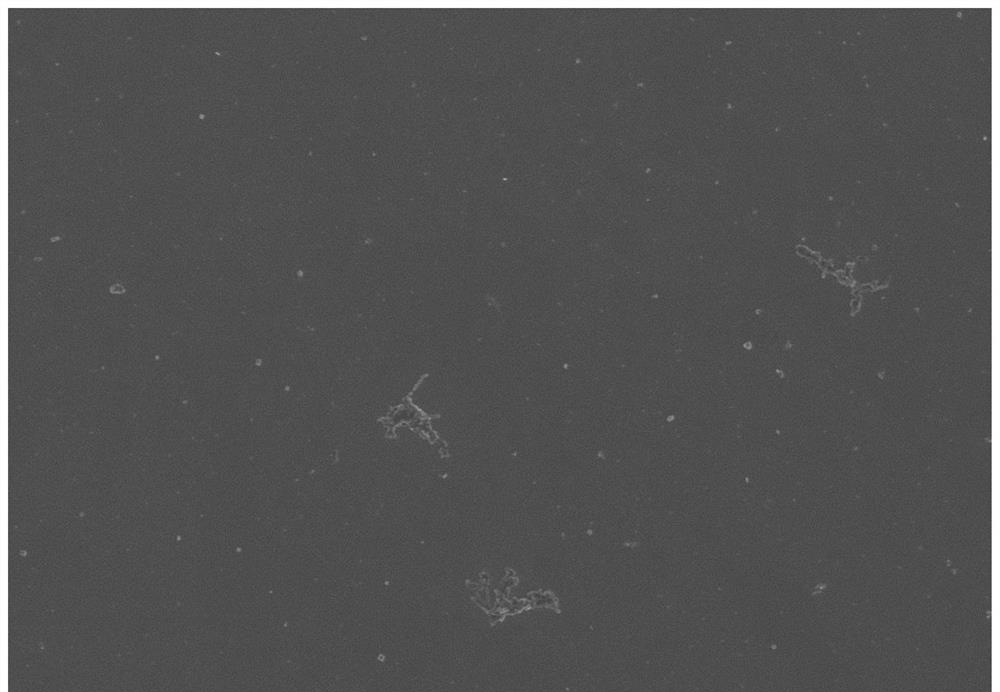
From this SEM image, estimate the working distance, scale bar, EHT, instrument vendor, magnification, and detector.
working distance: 9.1 mm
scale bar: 50000 nm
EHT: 5 kV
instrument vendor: Hitachi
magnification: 1 K X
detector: SE(UL)